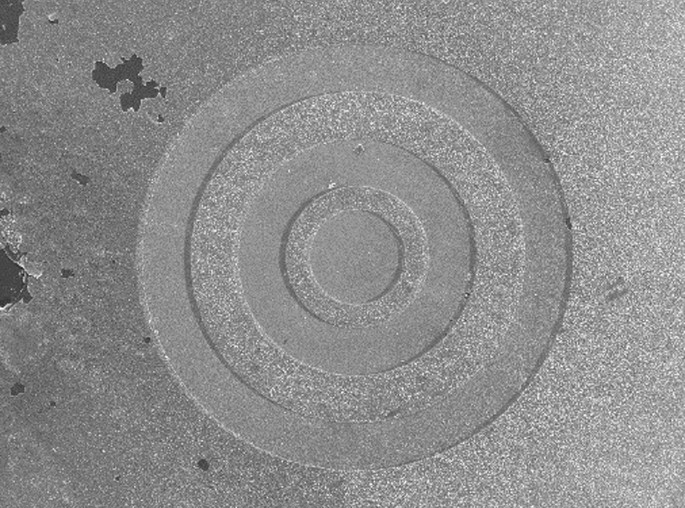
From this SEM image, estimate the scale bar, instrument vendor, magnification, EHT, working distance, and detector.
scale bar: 100000 nm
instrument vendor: JEOL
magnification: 0.085 K X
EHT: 15 kV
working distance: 10.4 mm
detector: SED